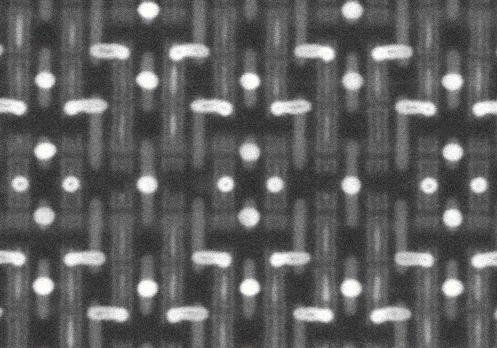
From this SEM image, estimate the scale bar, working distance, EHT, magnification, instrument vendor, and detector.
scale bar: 200 nm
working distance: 7.1 mm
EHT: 30 kV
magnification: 250 K X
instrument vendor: Hitachi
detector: OCD